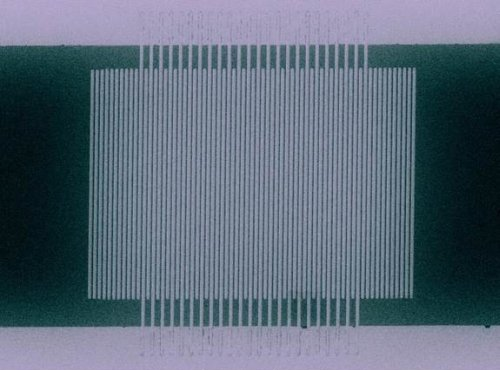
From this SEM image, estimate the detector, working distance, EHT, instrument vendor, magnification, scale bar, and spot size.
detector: SE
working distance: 5 mm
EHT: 30 kV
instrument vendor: FEI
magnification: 3.5 K X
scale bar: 5000 nm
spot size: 3